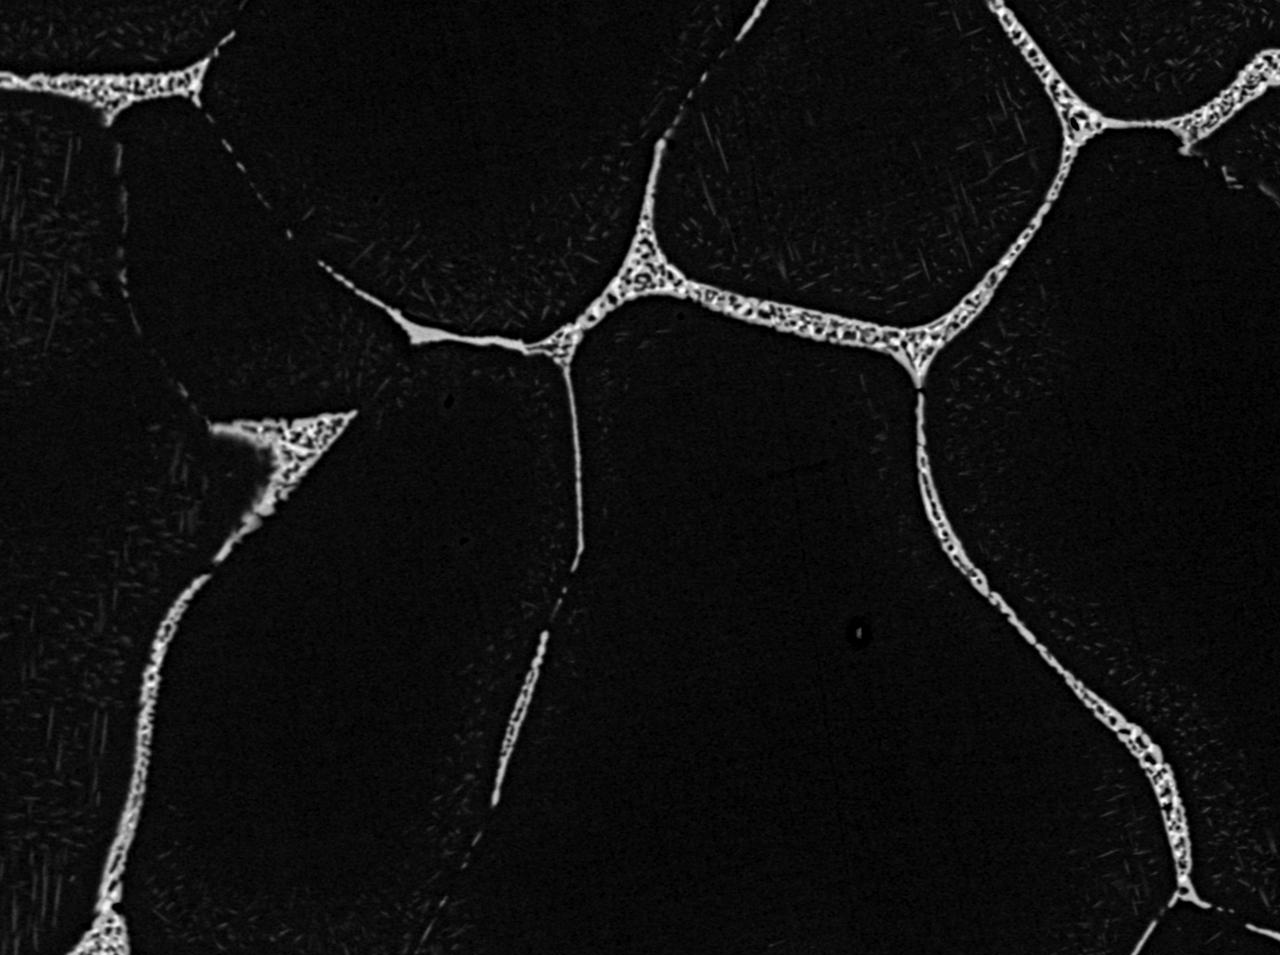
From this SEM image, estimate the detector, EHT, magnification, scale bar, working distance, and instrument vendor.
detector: BED-C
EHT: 20 kV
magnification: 0.5 K X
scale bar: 10000 nm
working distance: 8.9 mm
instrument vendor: JEOL